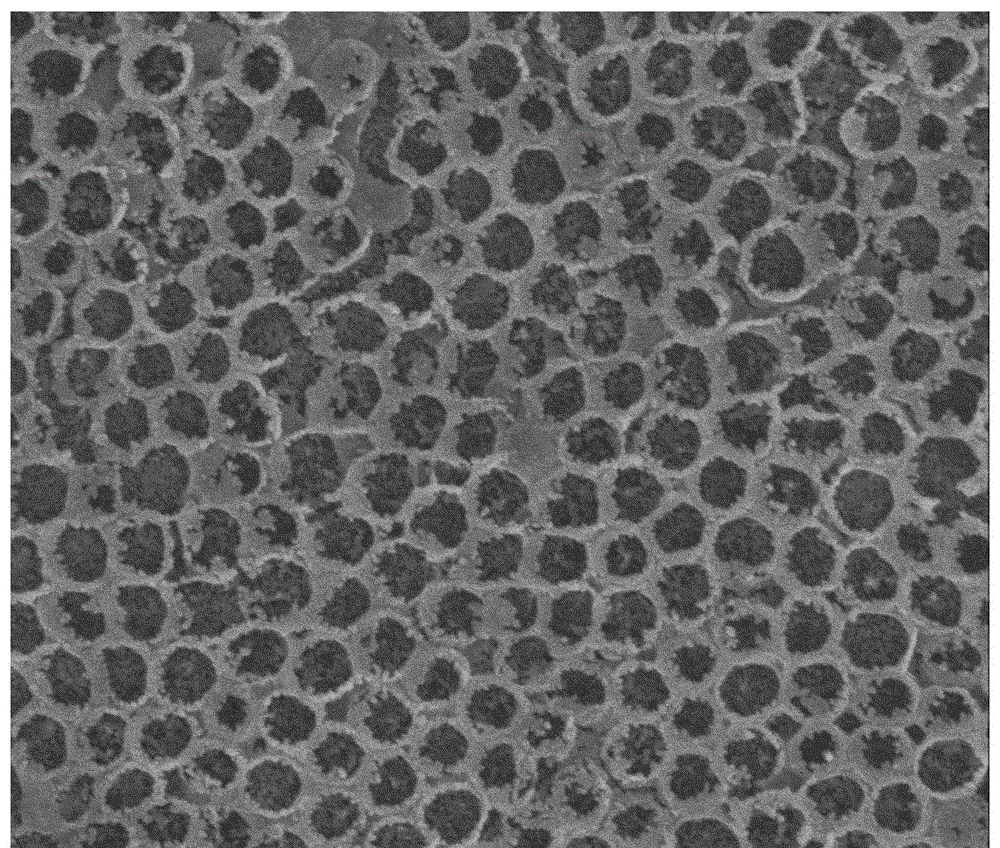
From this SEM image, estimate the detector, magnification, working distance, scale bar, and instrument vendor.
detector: TLD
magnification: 30 K X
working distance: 4.8 mm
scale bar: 2000 nm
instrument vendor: FEI